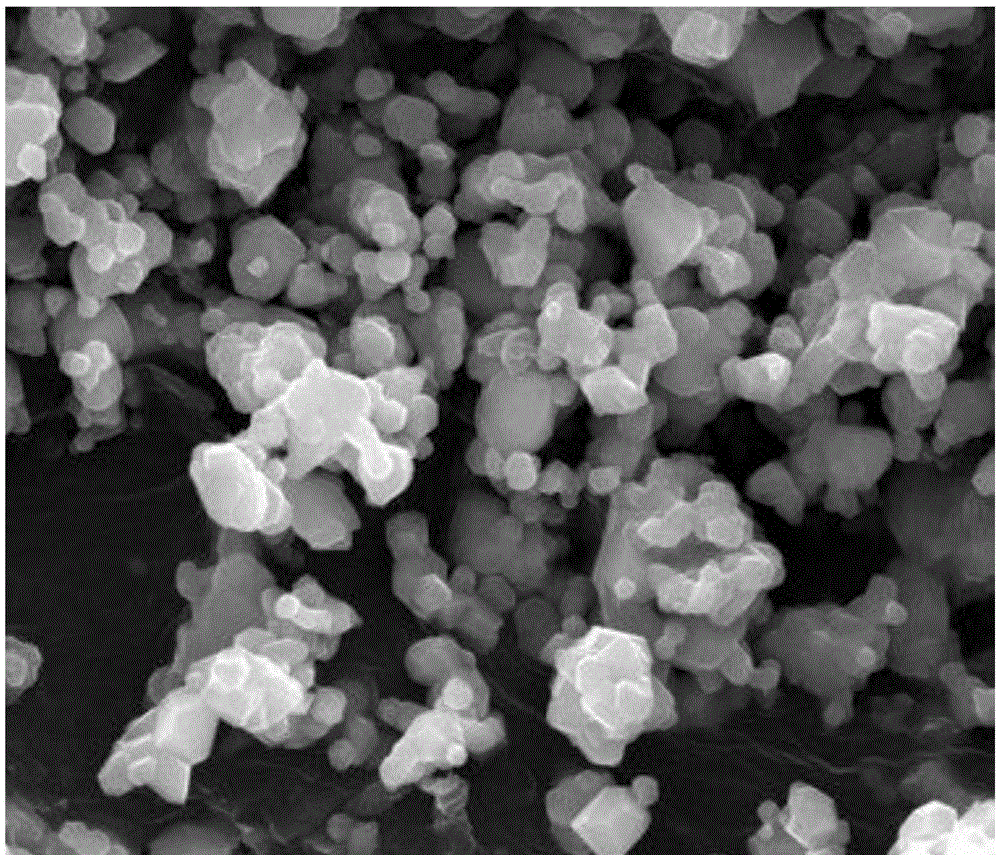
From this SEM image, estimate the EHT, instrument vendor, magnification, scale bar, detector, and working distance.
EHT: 20 kV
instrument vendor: FEI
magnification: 20 K X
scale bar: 5000 nm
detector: ETD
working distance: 9.8 mm